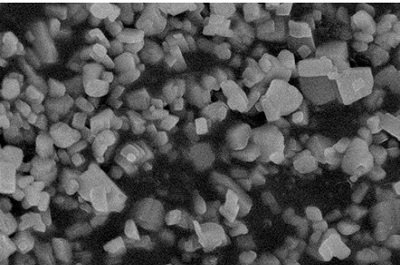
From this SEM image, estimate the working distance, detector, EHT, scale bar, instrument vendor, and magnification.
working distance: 8.3 mm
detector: SEI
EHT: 8 kV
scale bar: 1000 nm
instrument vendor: JEOL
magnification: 8 K X